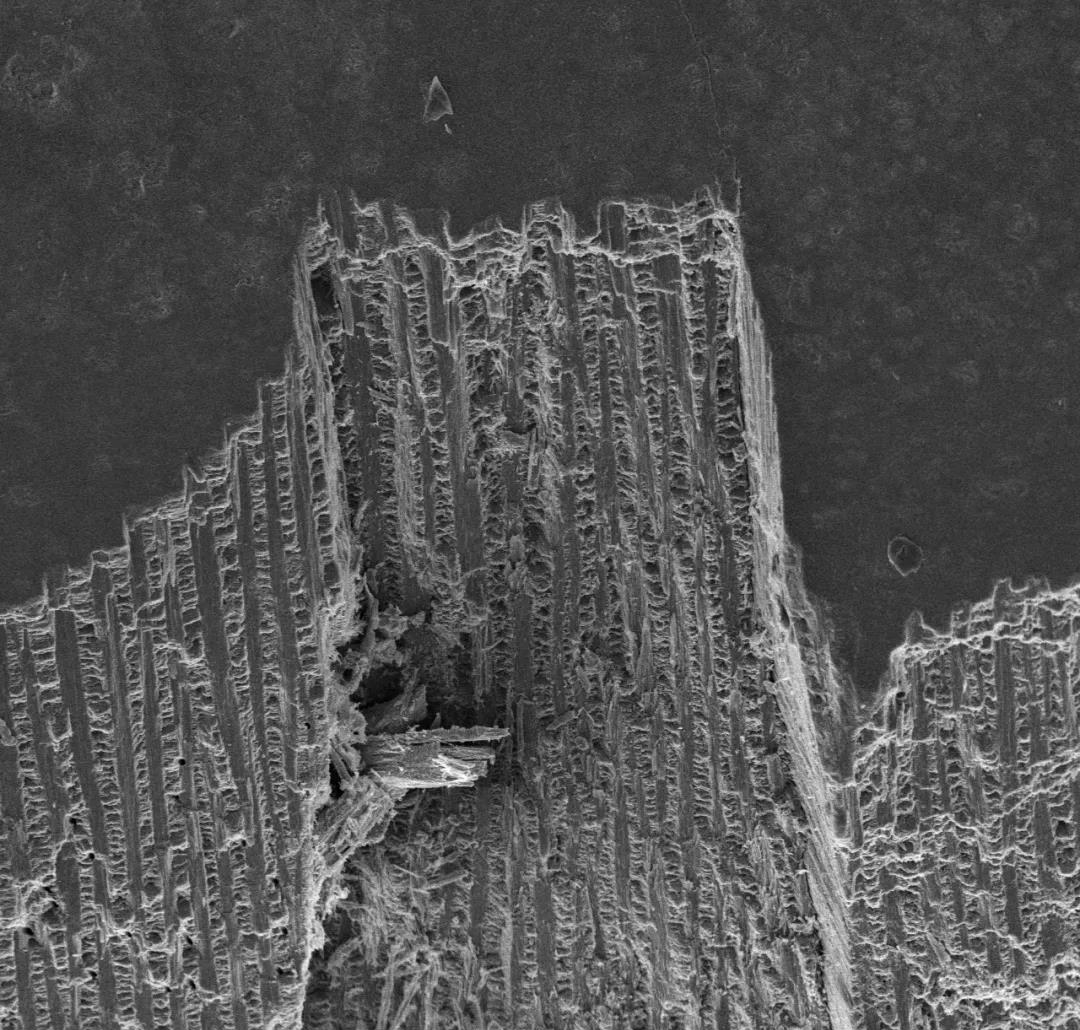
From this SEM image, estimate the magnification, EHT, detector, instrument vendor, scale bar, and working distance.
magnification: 0.5 K X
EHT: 15 kV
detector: SE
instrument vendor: other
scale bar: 100000 nm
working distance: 9.1 mm